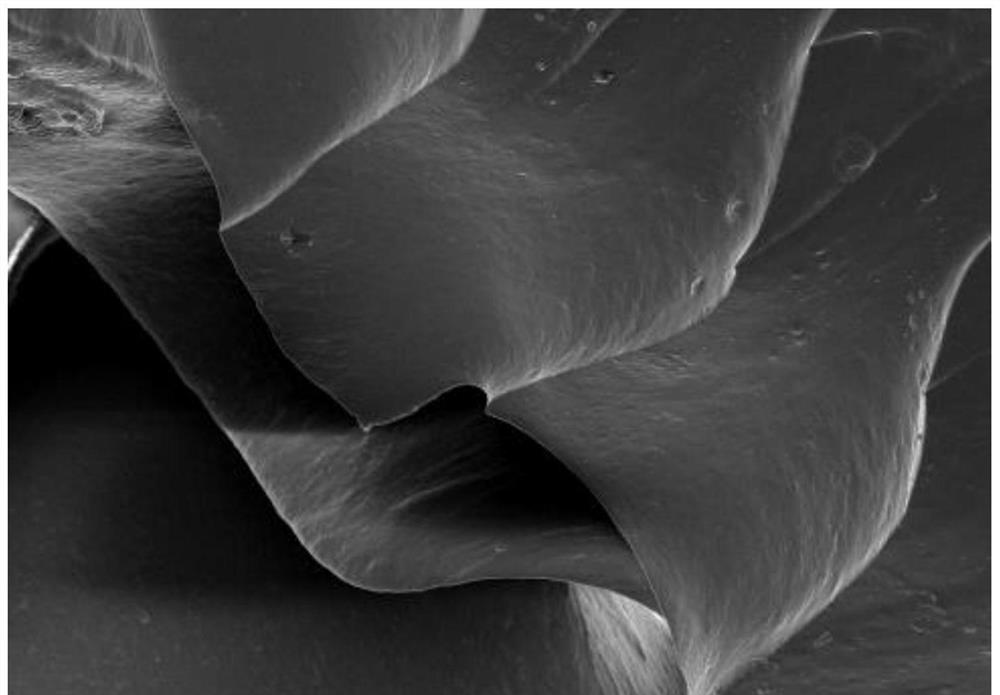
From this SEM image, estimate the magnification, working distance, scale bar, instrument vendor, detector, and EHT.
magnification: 0.5 K X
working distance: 14 mm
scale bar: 100000 nm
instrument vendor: Hitachi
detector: SE(U)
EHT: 5 kV